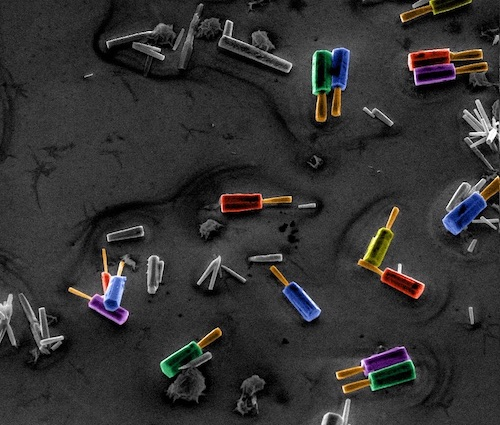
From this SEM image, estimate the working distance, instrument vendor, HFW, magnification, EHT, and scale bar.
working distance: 12.5 mm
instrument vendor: FEI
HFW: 43.91 µm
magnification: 7.288 K X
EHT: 10 kV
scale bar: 10000 nm